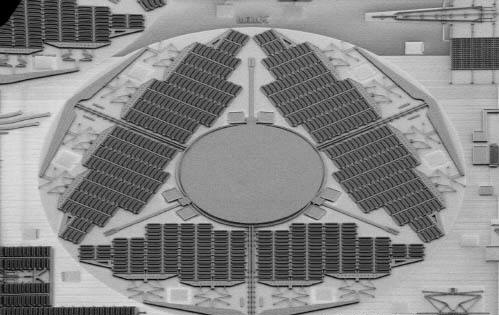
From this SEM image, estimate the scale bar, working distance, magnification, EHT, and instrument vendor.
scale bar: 1000000 nm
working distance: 13.7 mm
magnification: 0.035 K X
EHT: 0.8 kV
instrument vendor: Hitachi